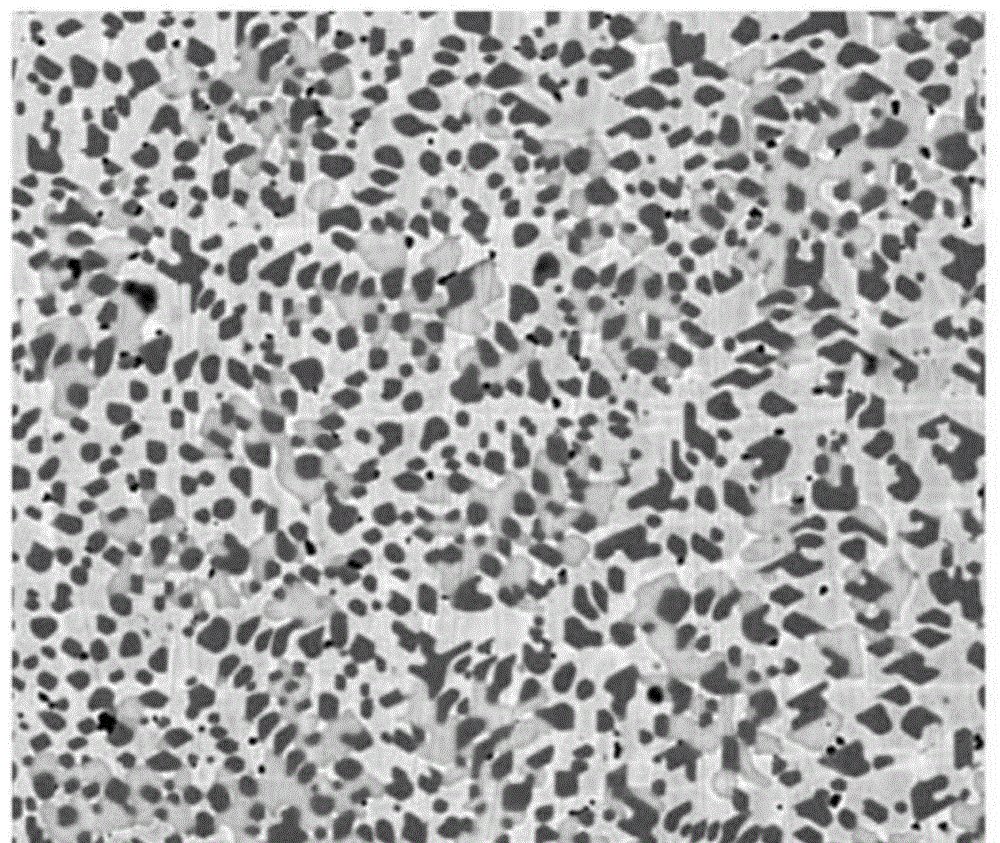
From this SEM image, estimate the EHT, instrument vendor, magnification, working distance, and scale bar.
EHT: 15 kV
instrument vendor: FEI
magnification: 5 K X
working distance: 6.7 mm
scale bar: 20000 nm